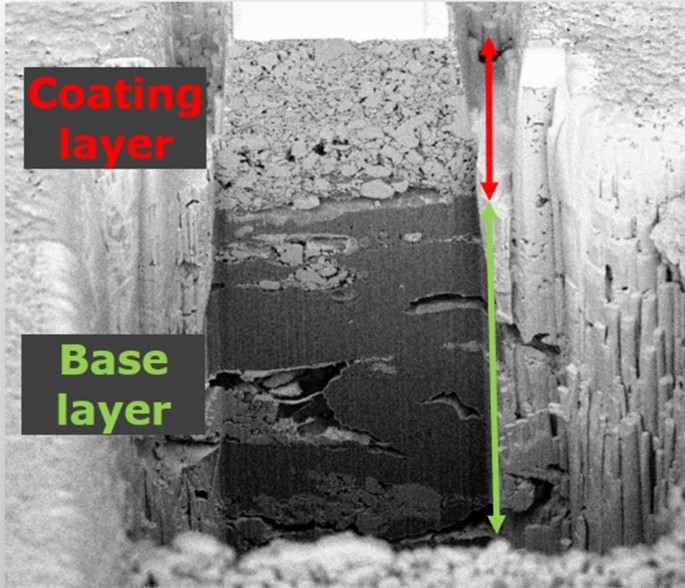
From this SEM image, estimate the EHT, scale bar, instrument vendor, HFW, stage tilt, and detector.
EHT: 2 kV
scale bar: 10000 nm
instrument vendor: FEI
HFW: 39.4 µm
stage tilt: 52°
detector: TLD